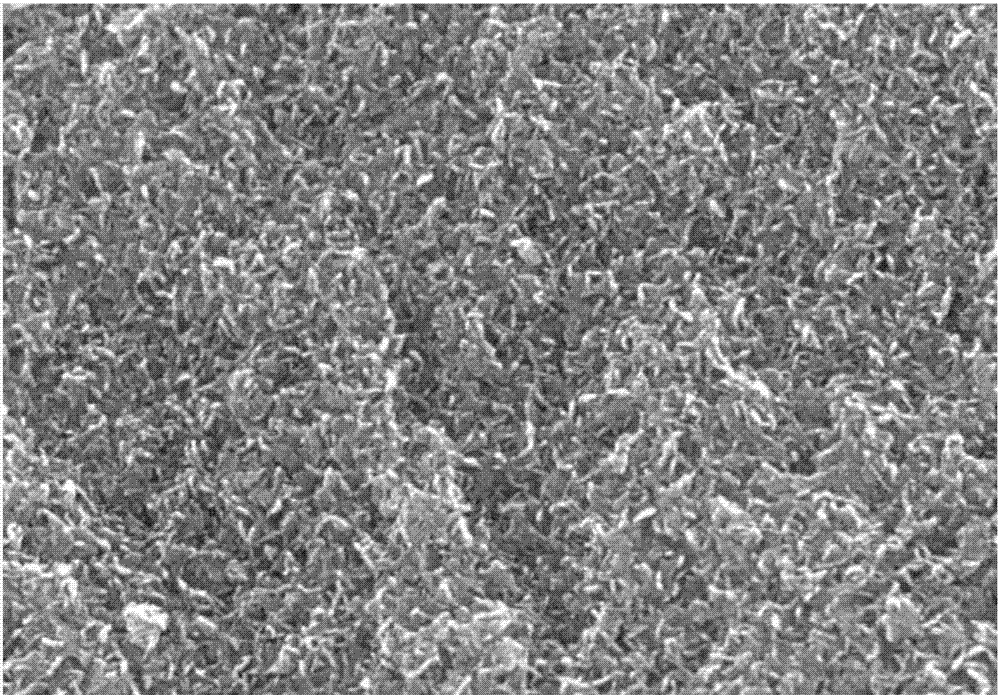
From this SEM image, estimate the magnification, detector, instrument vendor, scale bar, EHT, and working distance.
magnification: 80 K X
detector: SE(UL)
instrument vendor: Hitachi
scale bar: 500 nm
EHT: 15 kV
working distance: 8.7 mm